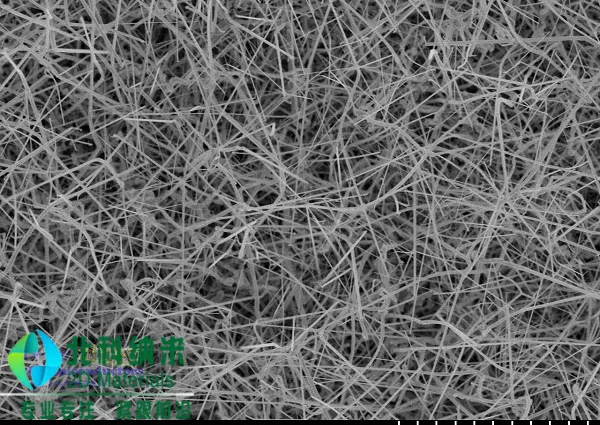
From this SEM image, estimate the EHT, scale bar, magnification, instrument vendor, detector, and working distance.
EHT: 5 kV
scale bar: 5000 nm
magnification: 8 K X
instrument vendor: Hitachi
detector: SE(M)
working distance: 7.8 mm